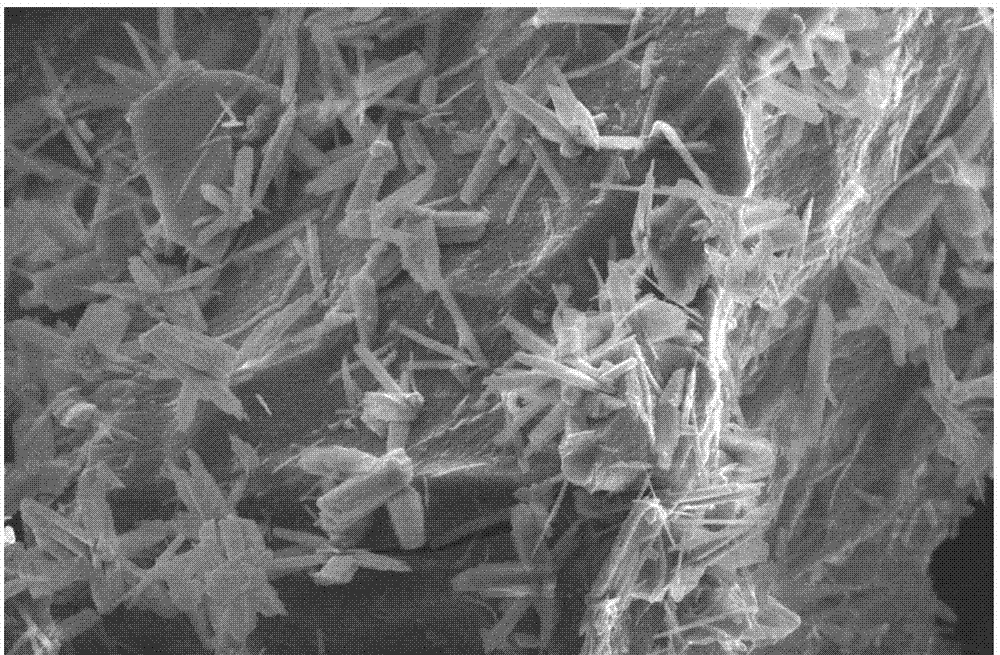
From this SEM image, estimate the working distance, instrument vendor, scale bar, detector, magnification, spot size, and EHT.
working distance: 4.9 mm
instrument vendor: FEI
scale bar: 2000 nm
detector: TLD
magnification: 20 K X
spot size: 3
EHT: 5 kV